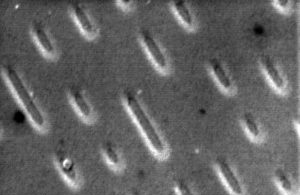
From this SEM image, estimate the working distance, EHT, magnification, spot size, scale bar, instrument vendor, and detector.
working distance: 17.4 mm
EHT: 5 kV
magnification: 7.586 K X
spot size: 4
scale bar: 2000 nm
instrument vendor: FEI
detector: SE